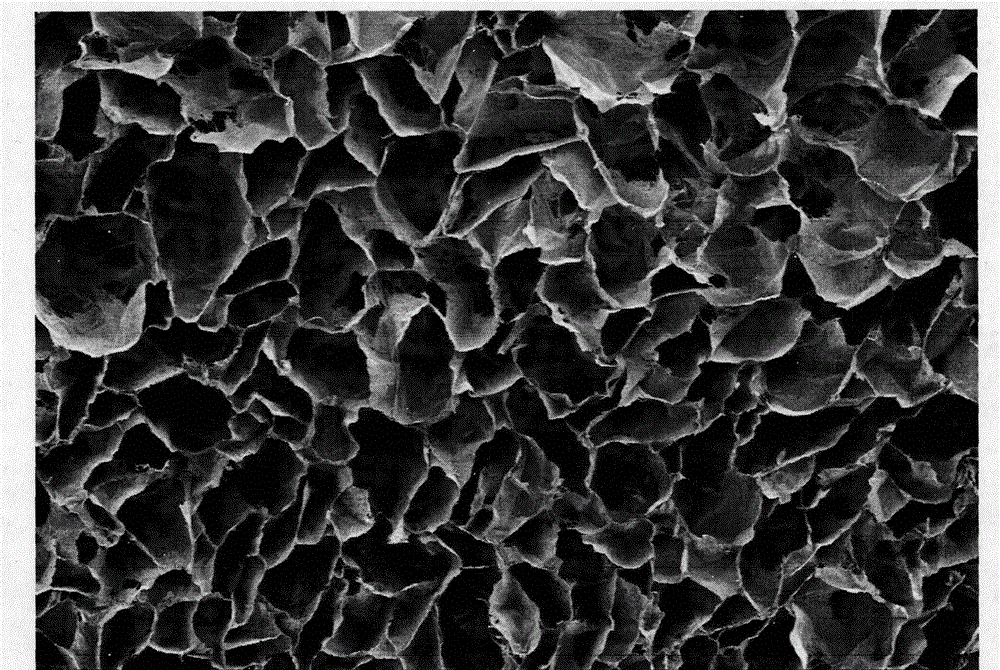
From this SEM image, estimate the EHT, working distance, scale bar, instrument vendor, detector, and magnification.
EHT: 5 kV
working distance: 13.5 mm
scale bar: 1000000 nm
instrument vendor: Hitachi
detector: SE(M)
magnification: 0.05 K X